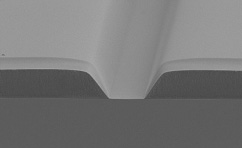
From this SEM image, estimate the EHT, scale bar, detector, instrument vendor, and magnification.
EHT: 3 kV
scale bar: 10000 nm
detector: SE(M)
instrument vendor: Hitachi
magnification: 4 K X